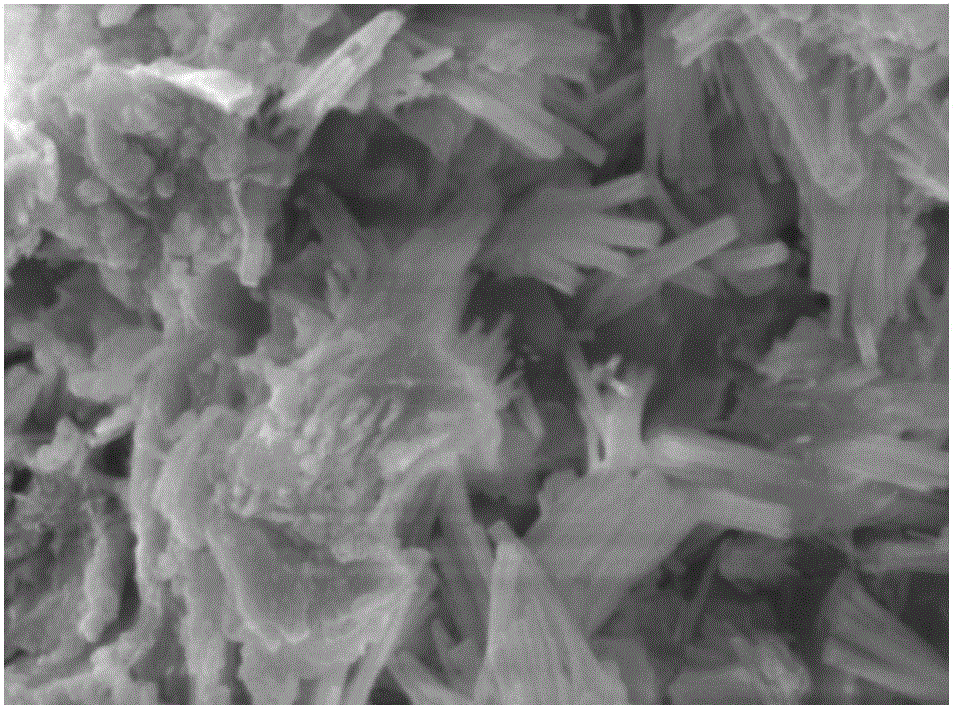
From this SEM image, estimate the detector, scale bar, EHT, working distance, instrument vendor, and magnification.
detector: SEI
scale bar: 1000 nm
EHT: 15 kV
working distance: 10 mm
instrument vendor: JEOL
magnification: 5 K X